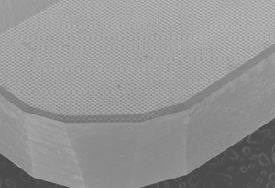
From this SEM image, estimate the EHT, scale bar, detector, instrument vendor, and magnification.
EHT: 15 kV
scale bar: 1000000 nm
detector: SEI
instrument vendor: JEOL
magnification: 0.02 K X